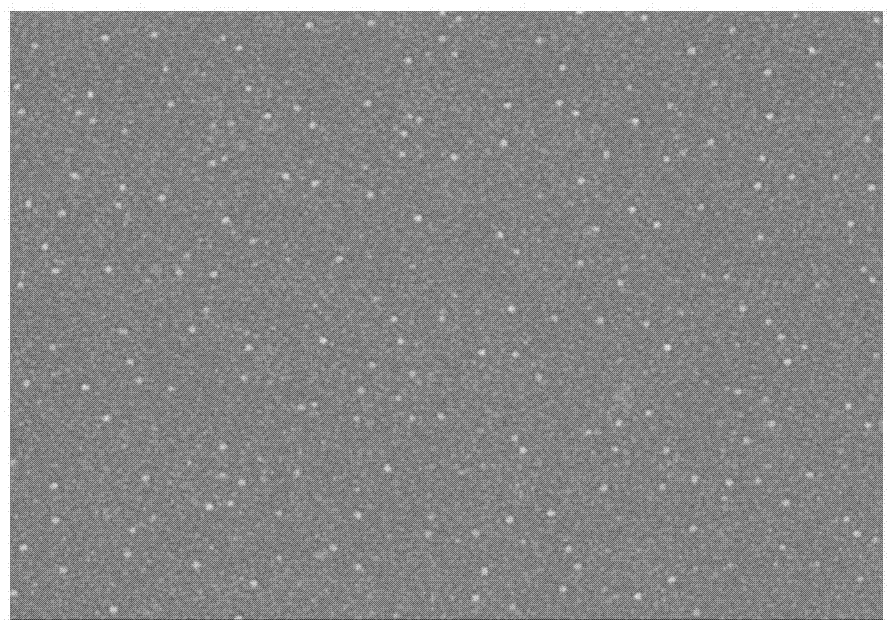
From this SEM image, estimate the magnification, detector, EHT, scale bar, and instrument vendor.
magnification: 30 K X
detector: SE(M)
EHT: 8 kV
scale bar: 1000 nm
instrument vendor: Hitachi